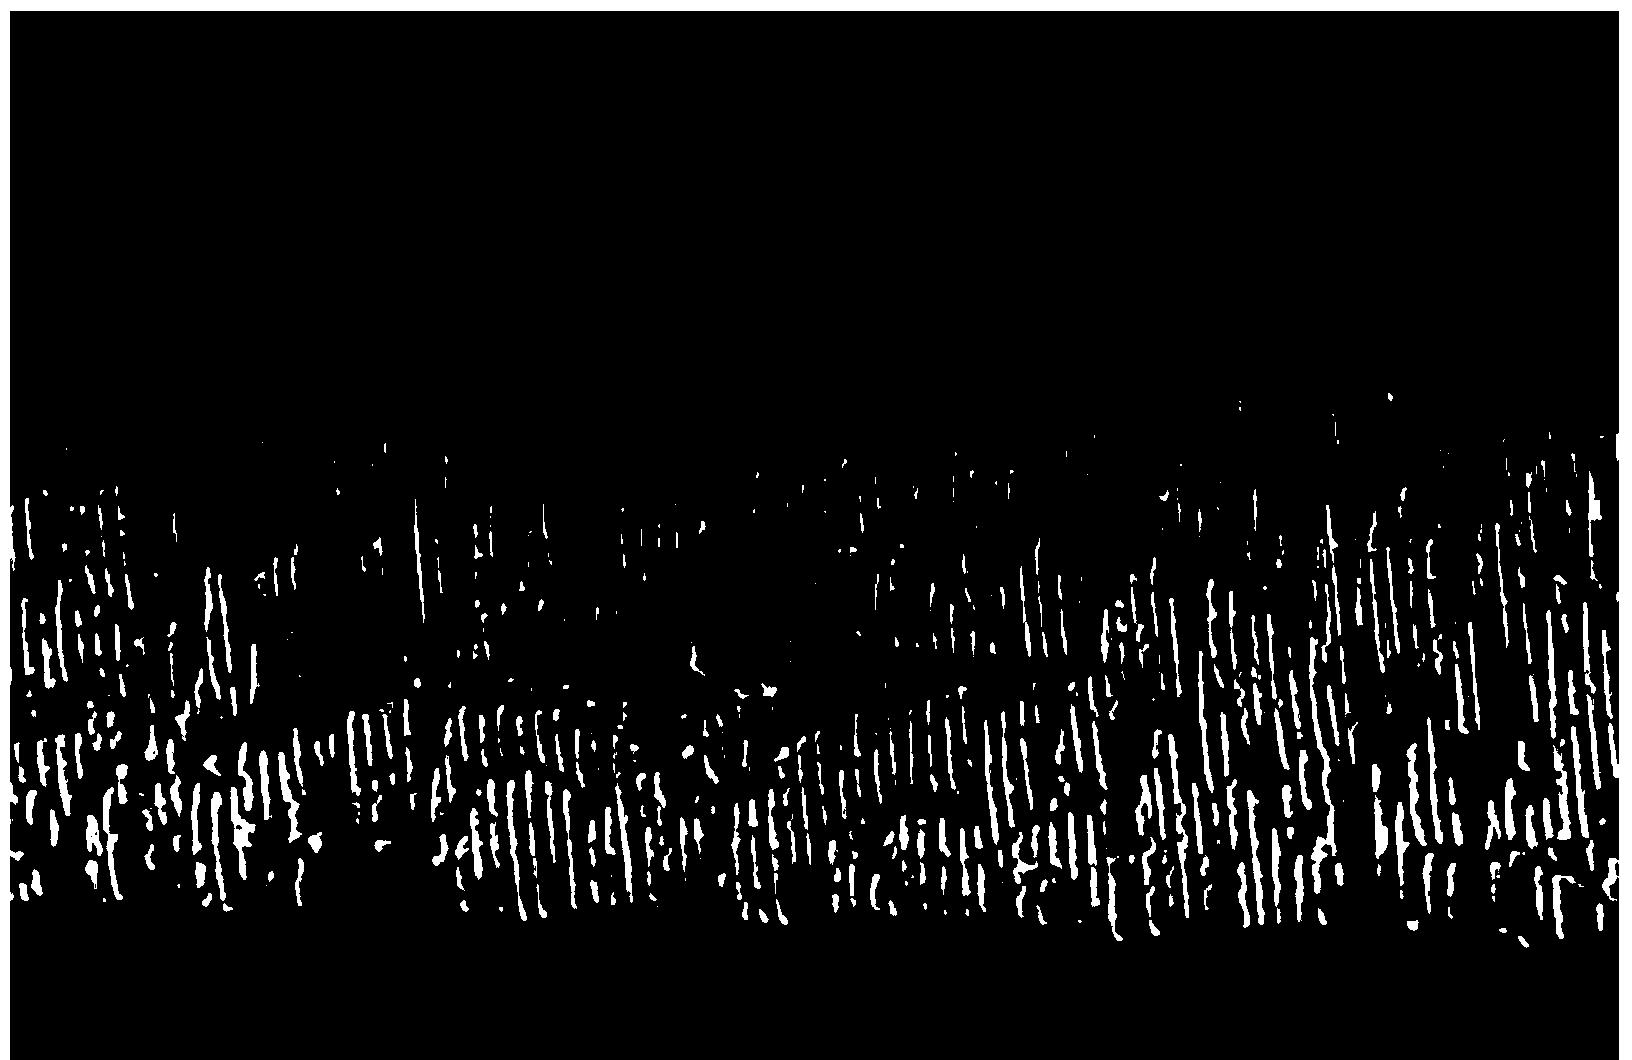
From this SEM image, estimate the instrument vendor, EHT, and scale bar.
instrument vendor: JEOL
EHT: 10 kV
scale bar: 50000 nm